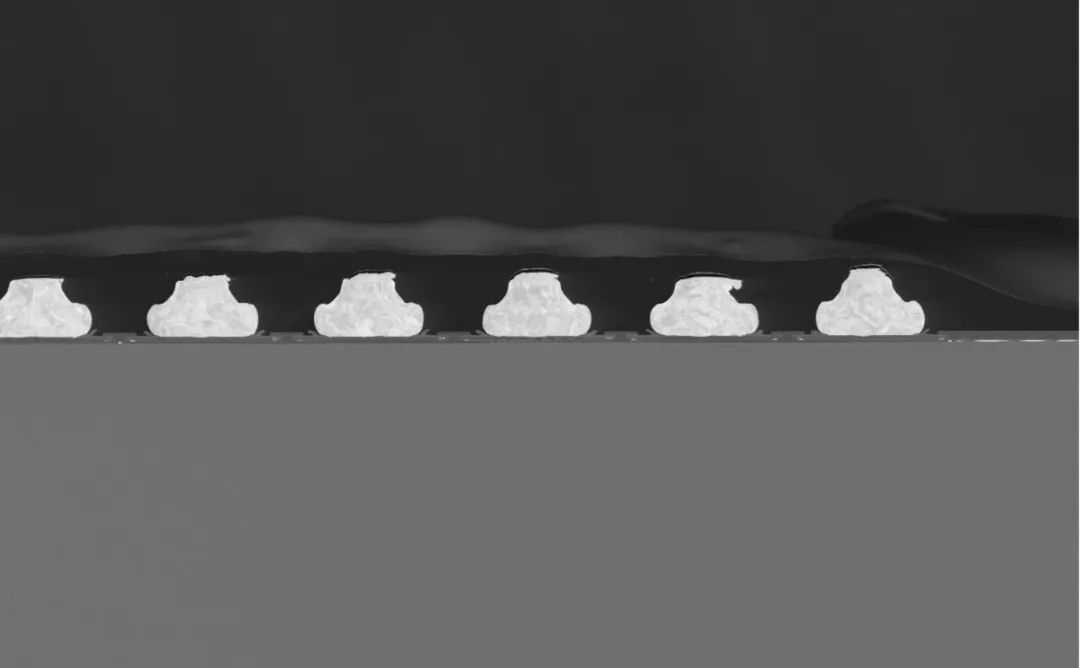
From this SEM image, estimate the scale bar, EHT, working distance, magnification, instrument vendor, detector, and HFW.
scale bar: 150000 nm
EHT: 5 kV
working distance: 7.484 mm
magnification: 1 K X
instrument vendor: other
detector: BSD Full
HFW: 519 µm